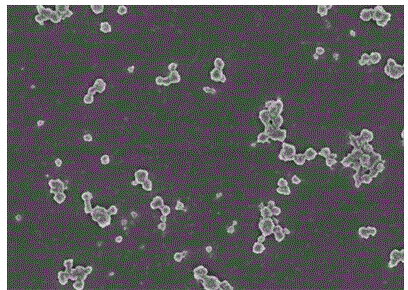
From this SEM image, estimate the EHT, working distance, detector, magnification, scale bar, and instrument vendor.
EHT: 3 kV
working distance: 10.3 mm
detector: SE(U)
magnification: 5 K X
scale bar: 10000 nm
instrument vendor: Hitachi